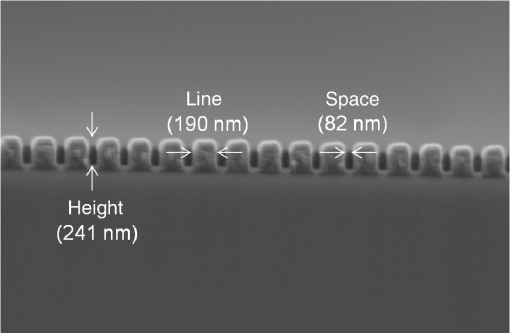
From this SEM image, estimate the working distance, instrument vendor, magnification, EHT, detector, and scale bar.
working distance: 14.4 mm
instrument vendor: Zeiss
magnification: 26.57 K X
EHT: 5 kV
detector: InLens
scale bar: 200 nm